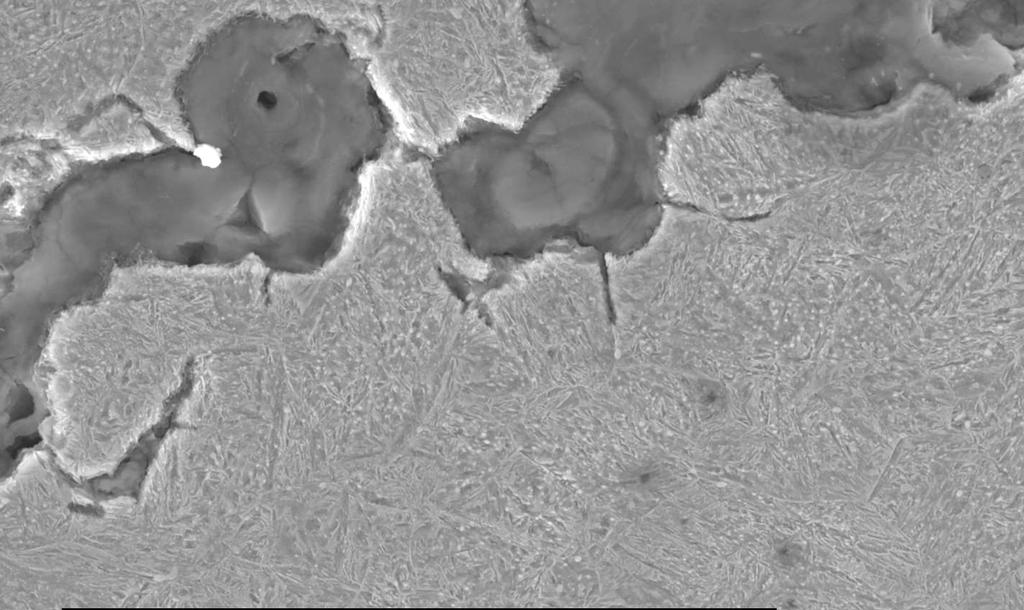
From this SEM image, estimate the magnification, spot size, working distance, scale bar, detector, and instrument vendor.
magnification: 1.5 K X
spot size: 5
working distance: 11 mm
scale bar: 20000 nm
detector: SE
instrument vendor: FEI